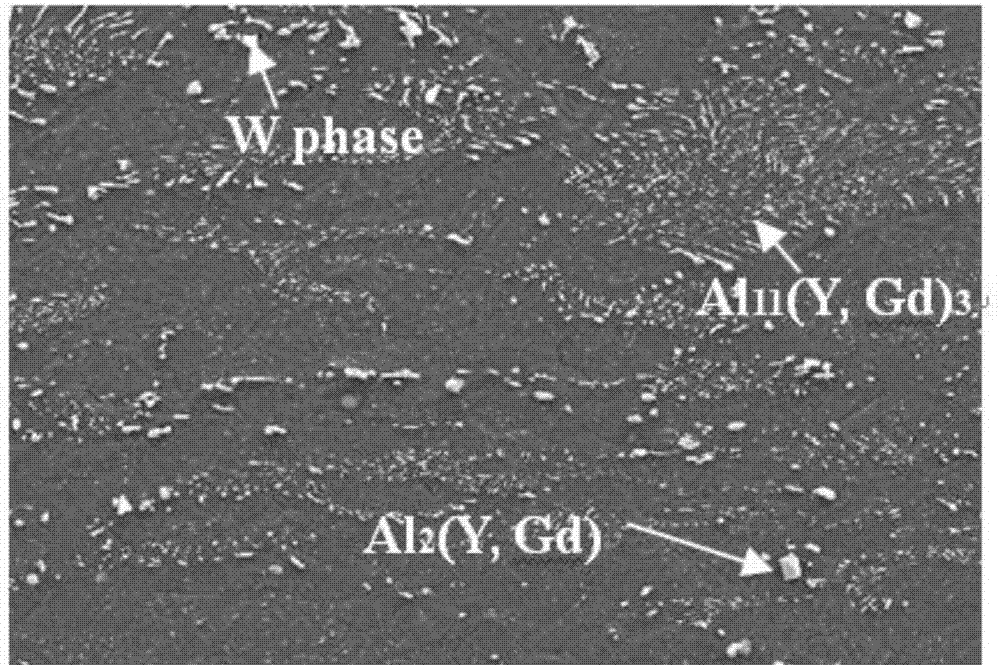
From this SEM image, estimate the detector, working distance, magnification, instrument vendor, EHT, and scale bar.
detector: SE2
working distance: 9 mm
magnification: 1 K X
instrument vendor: Zeiss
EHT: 15 kV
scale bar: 10000 nm